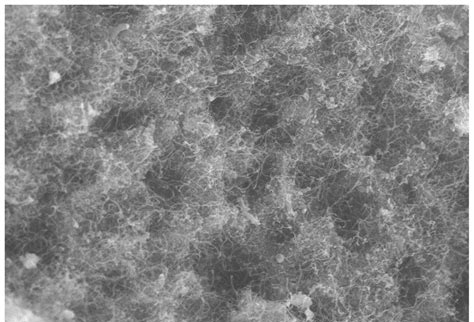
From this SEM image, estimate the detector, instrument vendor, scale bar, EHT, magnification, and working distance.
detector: SE(UL)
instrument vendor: Hitachi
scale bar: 10000 nm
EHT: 15 kV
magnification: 4 K X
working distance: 8.8 mm